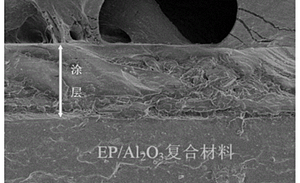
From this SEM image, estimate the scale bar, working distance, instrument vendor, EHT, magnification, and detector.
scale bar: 50000 nm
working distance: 11.2 mm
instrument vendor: Hitachi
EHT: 5 kV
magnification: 1 K X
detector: SE(M)